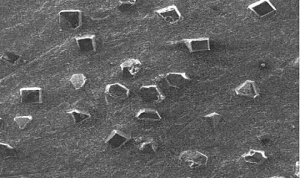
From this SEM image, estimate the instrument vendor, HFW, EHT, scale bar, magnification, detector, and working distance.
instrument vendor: FEI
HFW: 1280 µm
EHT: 20 kV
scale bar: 500000 nm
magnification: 0.2 K X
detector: ETD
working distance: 10.1 mm